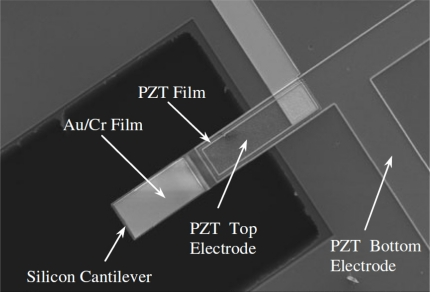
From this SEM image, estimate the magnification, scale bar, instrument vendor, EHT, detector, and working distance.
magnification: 0.25 K X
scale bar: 200000 nm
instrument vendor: Hitachi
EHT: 5 kV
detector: SE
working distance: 8.1 mm